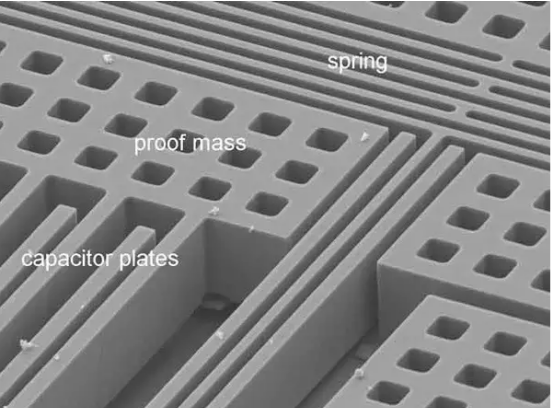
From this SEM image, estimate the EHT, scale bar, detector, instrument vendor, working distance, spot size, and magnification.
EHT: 10 kV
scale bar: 20000 nm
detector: SE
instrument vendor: FEI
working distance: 14.6 mm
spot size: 3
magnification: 1.5 K X